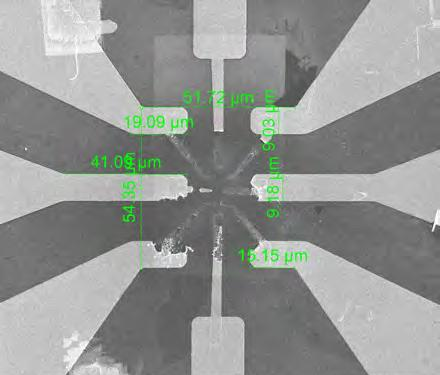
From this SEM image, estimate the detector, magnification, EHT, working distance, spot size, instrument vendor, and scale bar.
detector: ETD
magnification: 1 K X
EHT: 5 kV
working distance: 8.8 mm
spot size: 3.5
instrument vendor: FEI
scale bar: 50000 nm